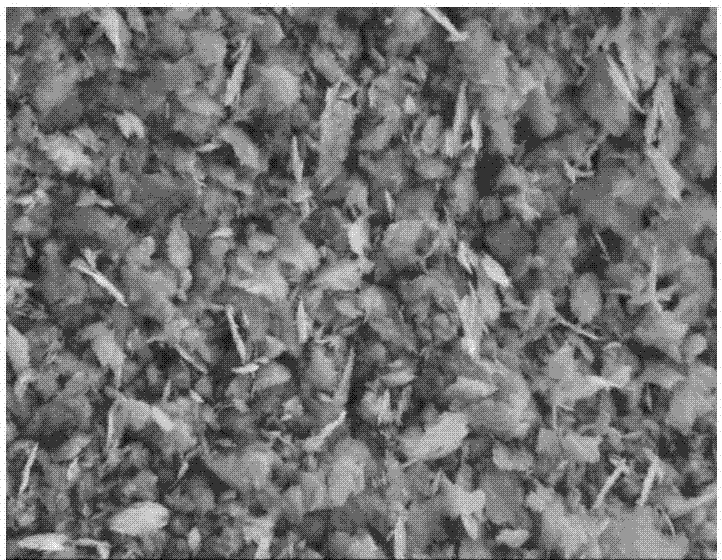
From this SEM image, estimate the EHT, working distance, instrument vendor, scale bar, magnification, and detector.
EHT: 15 kV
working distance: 14.9 mm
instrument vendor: Hitachi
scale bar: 20000 nm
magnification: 2 K X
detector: SE(M)